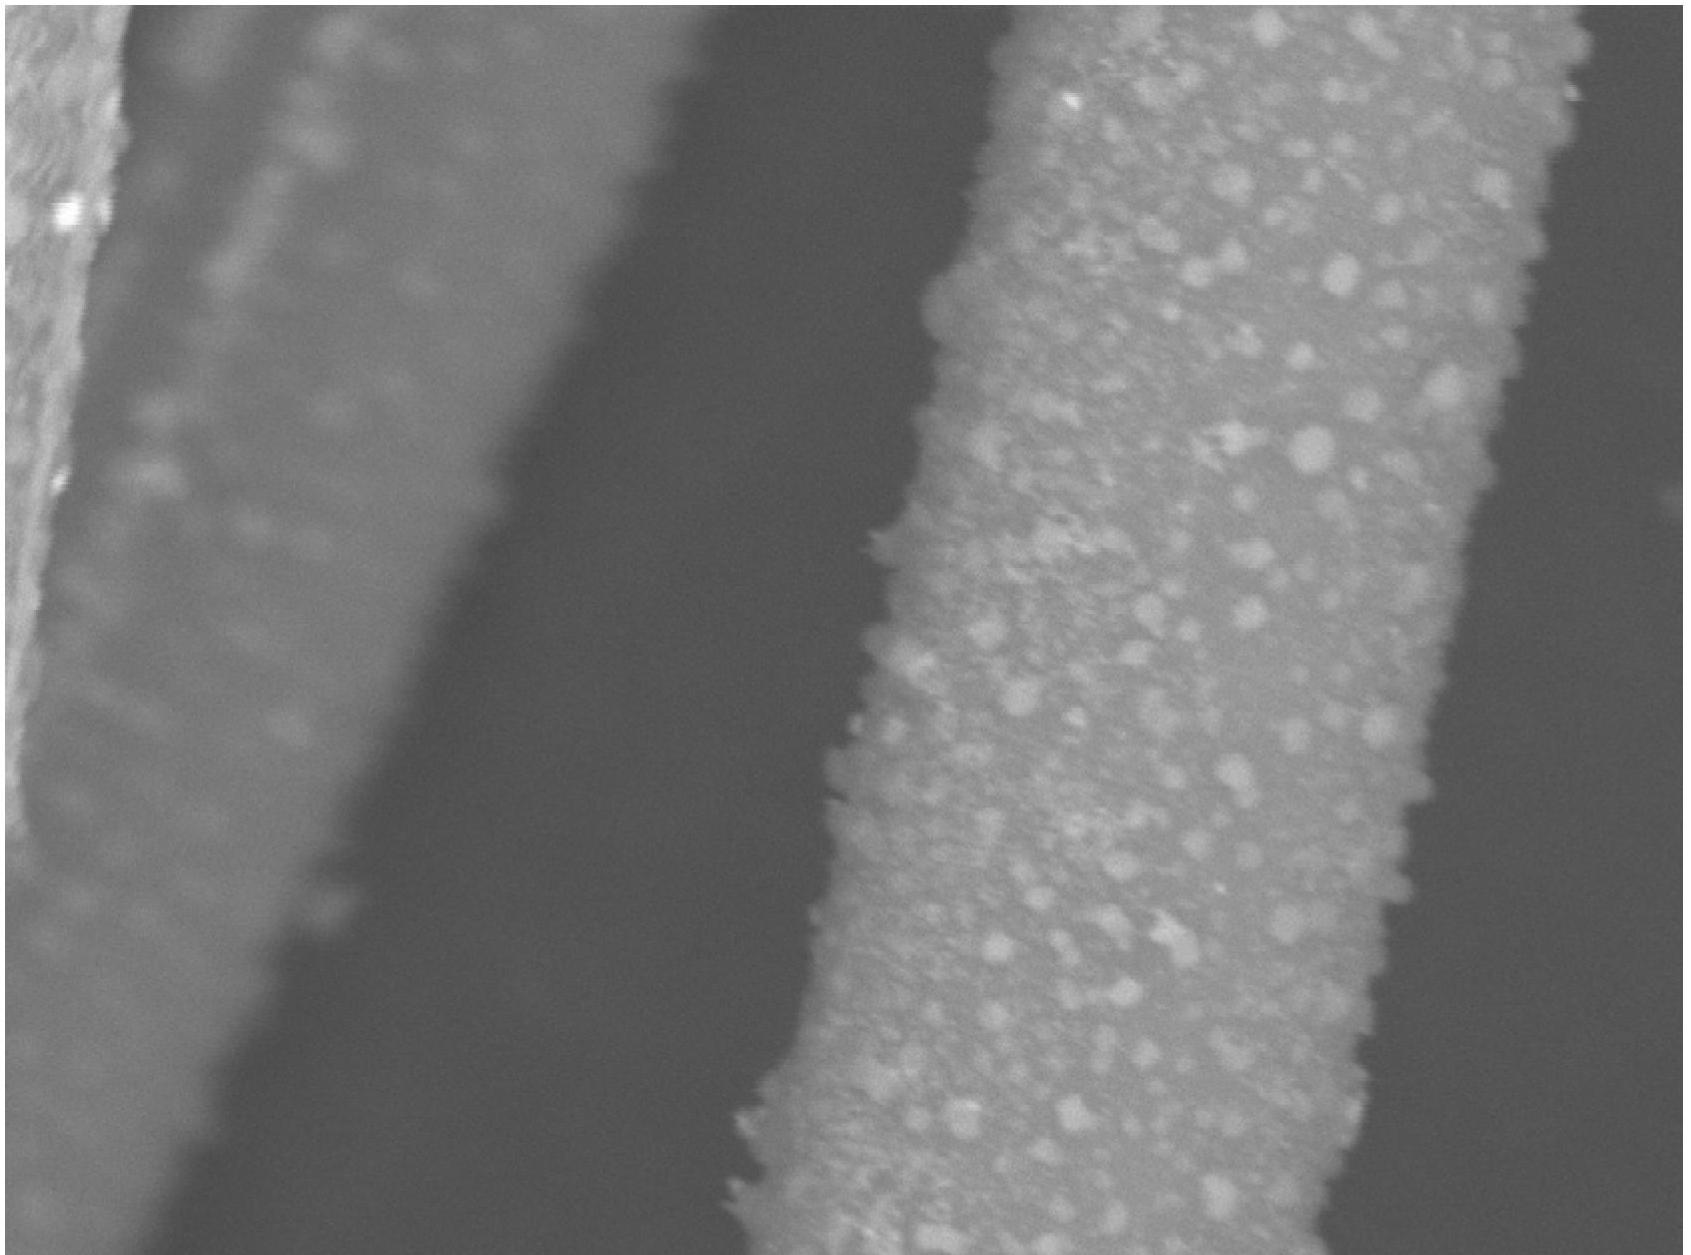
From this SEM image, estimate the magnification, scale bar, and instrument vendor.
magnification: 6 K X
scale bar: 10000 nm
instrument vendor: Hitachi